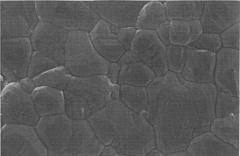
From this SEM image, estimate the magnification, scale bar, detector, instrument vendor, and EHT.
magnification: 2.7 K X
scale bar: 5000 nm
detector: SEI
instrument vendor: JEOL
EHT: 15 kV